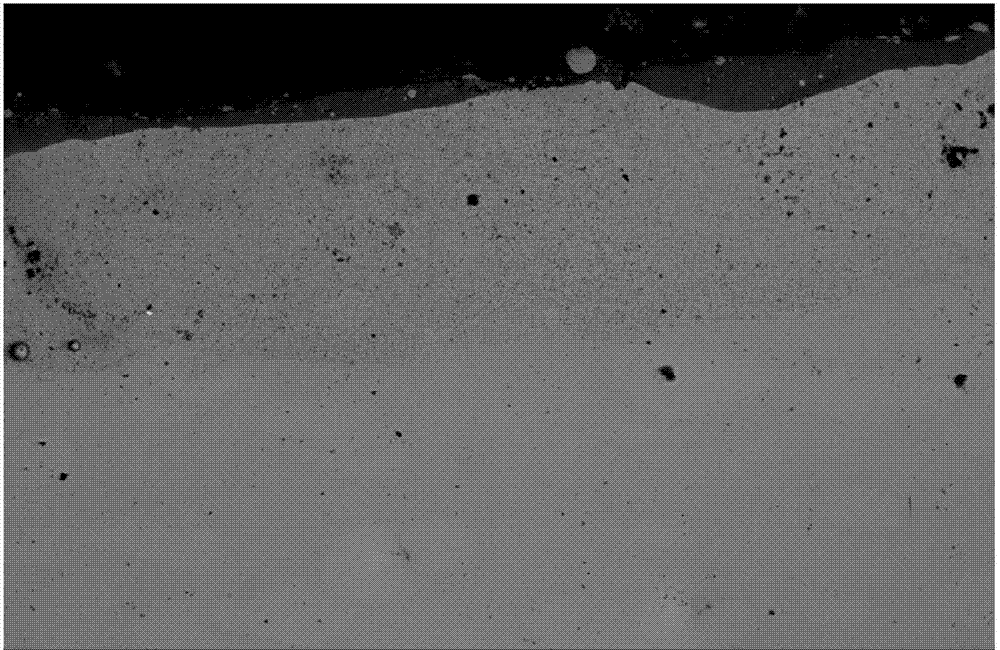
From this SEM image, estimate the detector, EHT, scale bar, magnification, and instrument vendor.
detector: BES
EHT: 20 kV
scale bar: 100000 nm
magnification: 0.1 K X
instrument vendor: JEOL